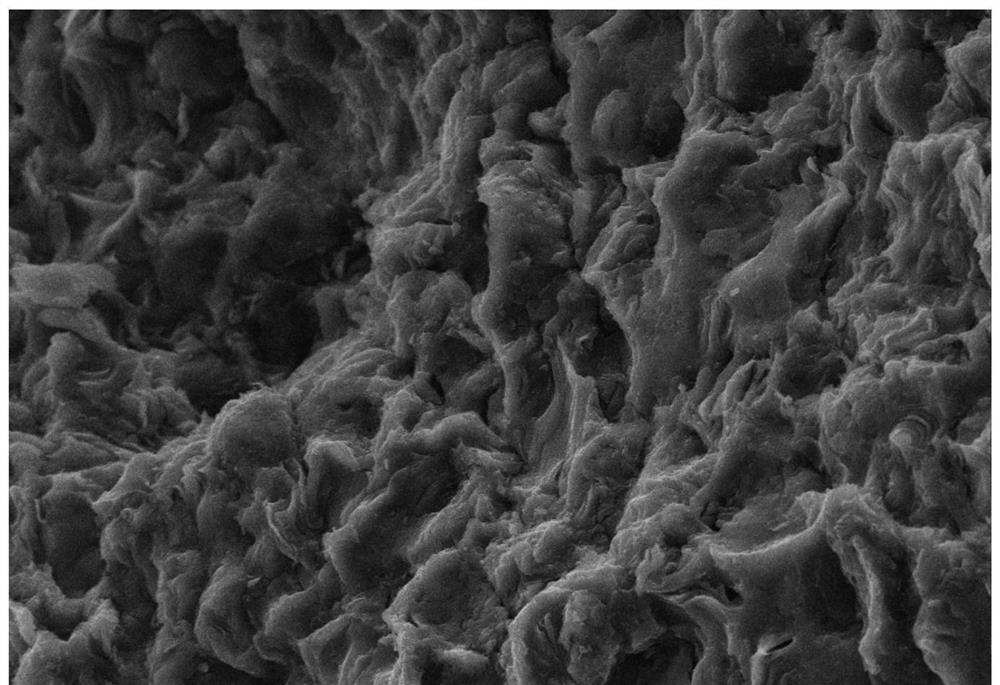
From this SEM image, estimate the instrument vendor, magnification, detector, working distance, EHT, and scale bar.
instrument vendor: other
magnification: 1 K X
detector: SE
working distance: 12.3 mm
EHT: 20 kV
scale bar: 20000 nm